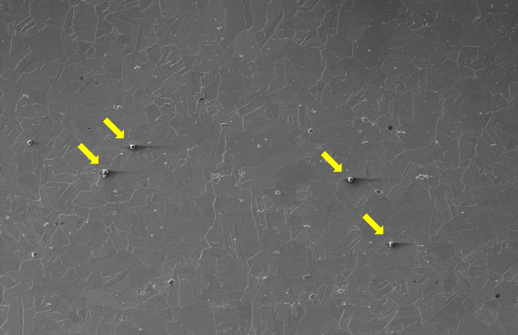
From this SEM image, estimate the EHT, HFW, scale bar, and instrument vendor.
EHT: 10 kV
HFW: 691 µm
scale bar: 200000 nm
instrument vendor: Thermo